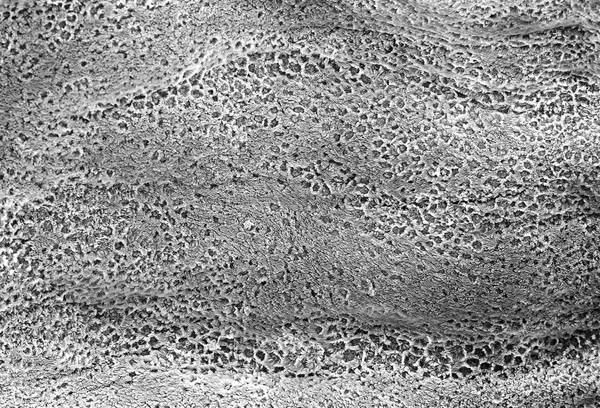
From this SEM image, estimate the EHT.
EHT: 10 kV